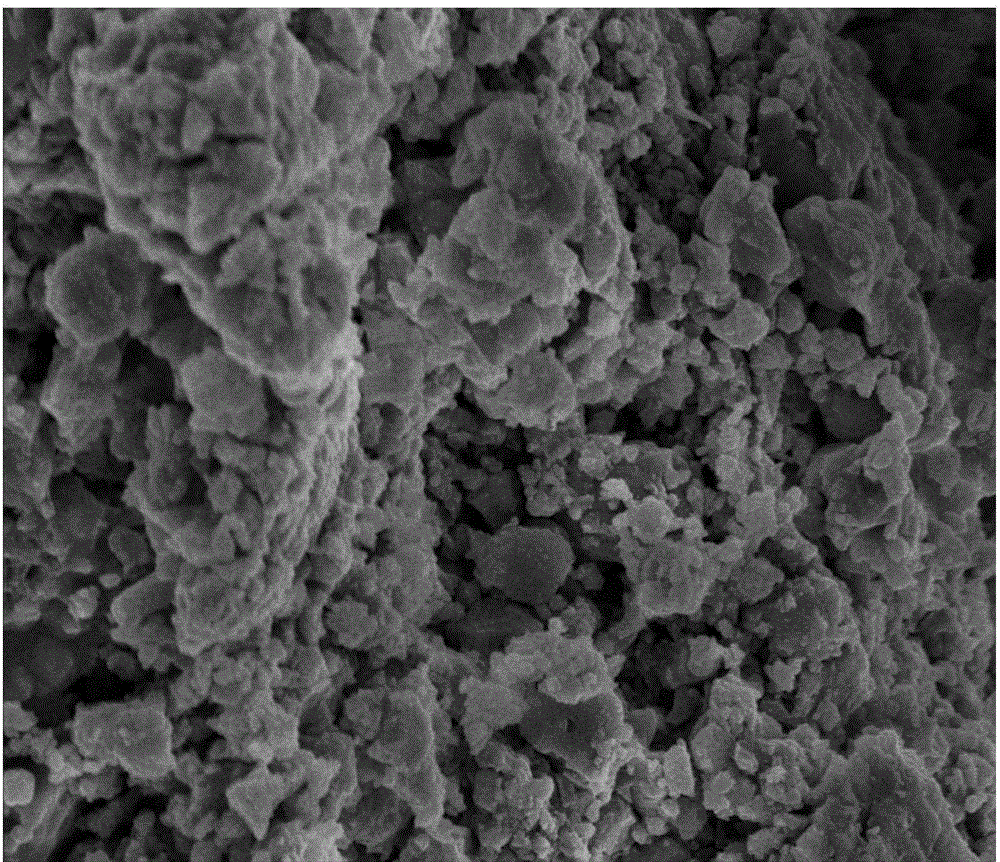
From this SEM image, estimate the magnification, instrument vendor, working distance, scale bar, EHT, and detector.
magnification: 20 K X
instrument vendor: FEI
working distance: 5.2 mm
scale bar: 2000 nm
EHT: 5 kV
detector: TLD